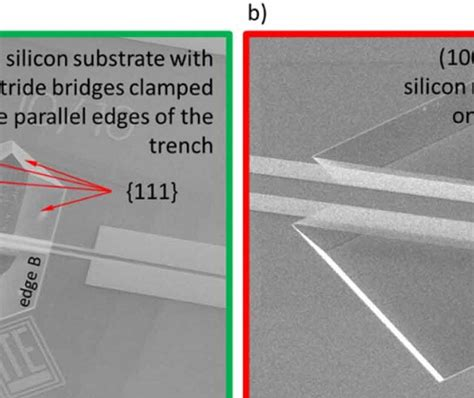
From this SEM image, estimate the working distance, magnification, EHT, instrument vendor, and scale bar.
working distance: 6.4 mm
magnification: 0.734 K X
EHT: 15 kV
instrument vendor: FEI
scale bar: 400000 nm